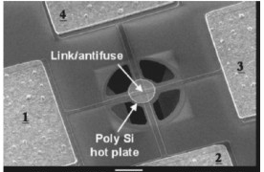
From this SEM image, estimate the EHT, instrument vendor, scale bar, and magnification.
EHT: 4 kV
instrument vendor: JEOL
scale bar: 10000 nm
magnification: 1.4 K X